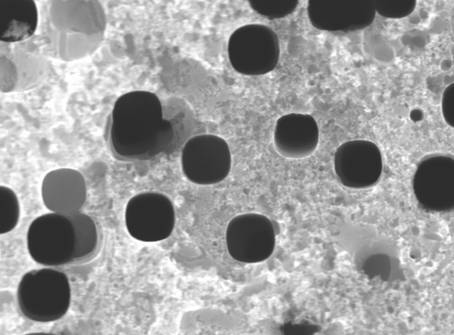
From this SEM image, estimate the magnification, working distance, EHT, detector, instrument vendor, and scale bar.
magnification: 30 K X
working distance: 6 mm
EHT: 15 kV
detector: SEI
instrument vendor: JEOL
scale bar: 100 nm